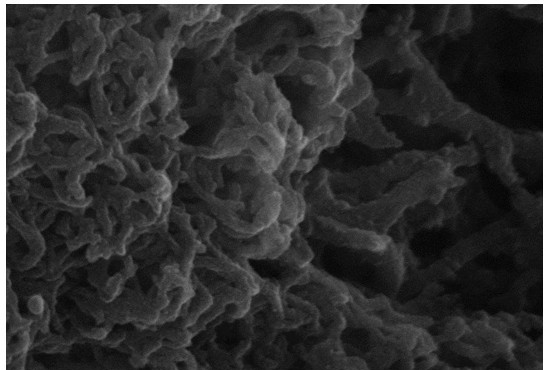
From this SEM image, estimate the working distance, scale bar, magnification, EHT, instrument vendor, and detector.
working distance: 8.5 mm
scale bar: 100 nm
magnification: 200 K X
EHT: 15 kV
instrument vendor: Zeiss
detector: InLens